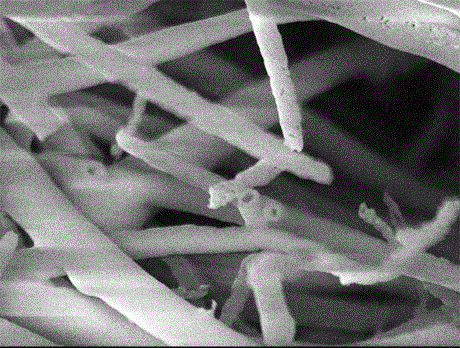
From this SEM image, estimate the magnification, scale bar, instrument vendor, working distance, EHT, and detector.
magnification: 20 K X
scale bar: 1000 nm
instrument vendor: JEOL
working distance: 7.9 mm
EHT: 5 kV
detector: SEI